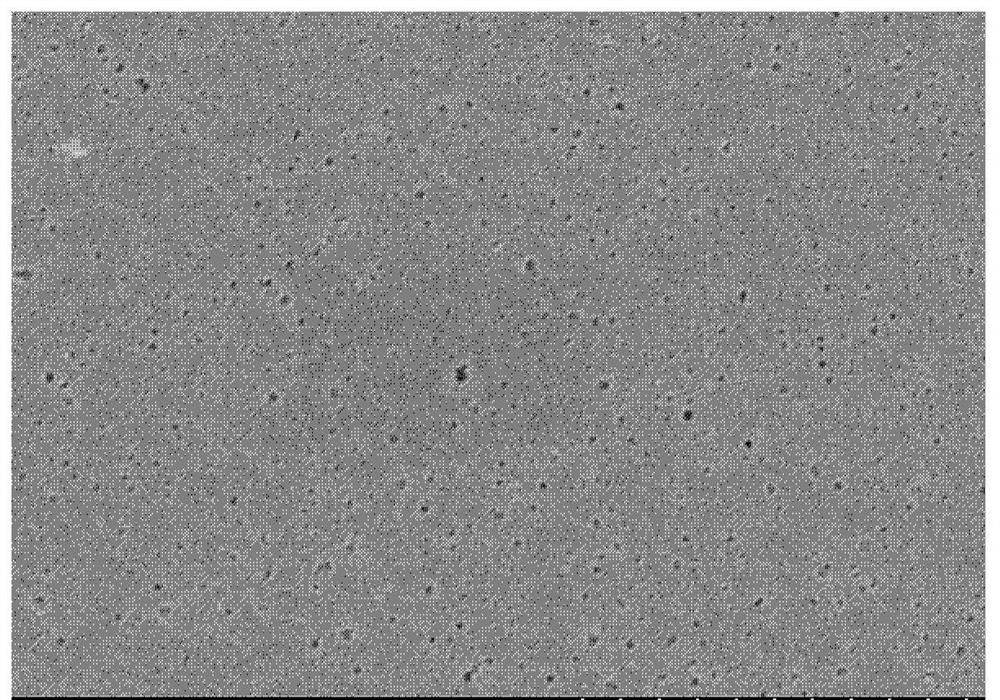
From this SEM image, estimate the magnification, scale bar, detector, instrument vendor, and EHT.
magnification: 50 K X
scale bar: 1000 nm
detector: SE(U)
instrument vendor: Hitachi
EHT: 5 kV